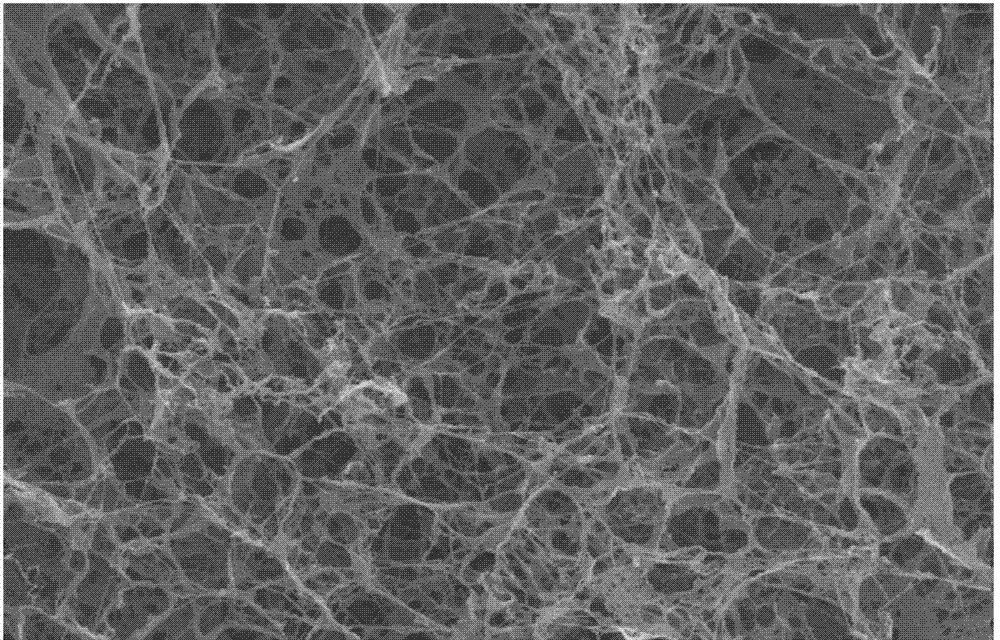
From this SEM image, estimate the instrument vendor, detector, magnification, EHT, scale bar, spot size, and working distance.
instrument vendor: FEI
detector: SE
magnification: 20 K X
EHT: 25 kV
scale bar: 2000 nm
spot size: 4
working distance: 6.9 mm